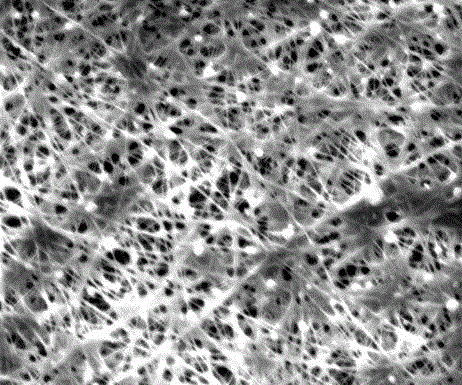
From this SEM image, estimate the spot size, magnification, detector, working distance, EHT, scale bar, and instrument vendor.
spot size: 3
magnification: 8 K X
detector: ETD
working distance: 14.4 mm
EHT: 10 kV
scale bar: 10000 nm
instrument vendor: FEI